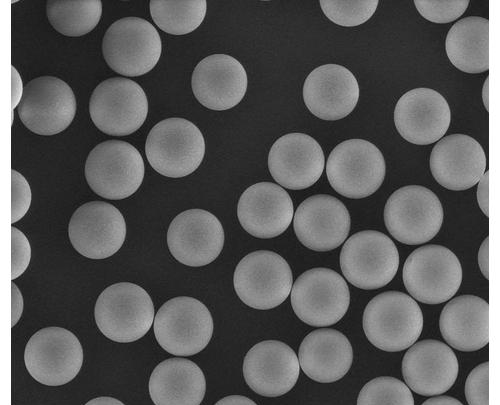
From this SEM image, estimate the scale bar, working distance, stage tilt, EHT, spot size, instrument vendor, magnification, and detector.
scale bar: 100000 nm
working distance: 12.3 mm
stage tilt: -0°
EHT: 20 kV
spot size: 3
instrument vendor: FEI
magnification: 0.76 K X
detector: LFD